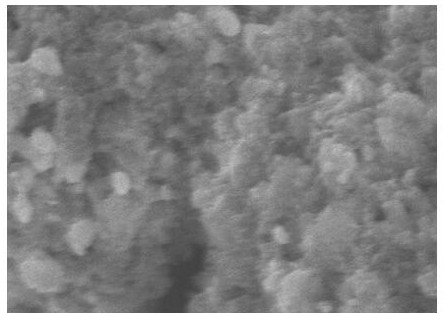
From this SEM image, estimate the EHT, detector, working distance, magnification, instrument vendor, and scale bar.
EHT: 5 kV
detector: SEI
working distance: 7.1 mm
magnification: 50 K X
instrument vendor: JEOL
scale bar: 100 nm